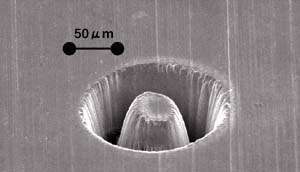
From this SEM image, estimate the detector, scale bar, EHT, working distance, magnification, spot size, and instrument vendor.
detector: SE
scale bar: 50000 nm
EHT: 25 kV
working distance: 11.6 mm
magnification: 0.4 K X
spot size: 3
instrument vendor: FEI